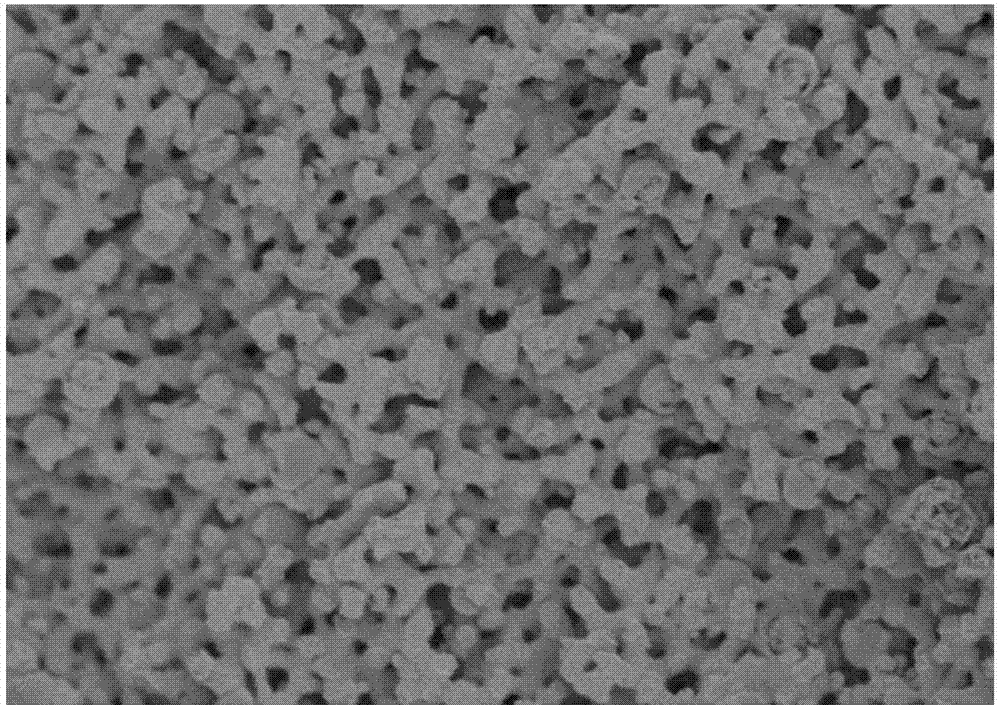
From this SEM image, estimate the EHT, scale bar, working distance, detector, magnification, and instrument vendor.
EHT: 3 kV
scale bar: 20000 nm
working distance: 8.5 mm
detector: SE(M)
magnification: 2 K X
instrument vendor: Hitachi